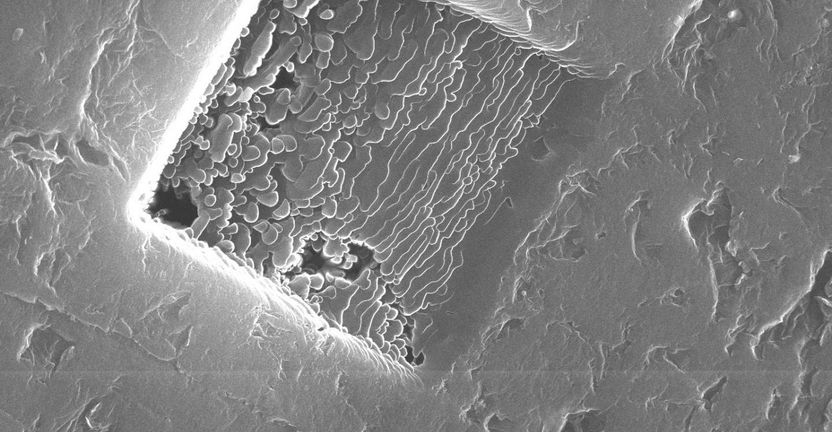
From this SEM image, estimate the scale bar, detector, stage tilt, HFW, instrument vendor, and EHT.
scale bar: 10000 nm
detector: SE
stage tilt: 0°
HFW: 34.5 µm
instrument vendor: FEI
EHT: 30 kV